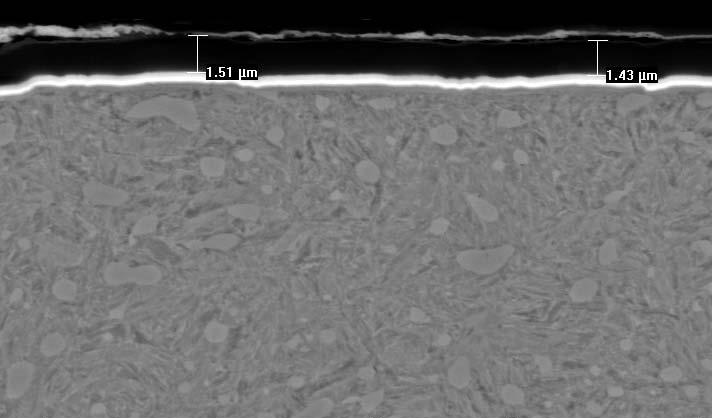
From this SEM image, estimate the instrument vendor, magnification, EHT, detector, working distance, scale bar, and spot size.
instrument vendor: FEI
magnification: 3.5 K X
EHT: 20 kV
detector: BSE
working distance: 9.2 mm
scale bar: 5000 nm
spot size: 3.5